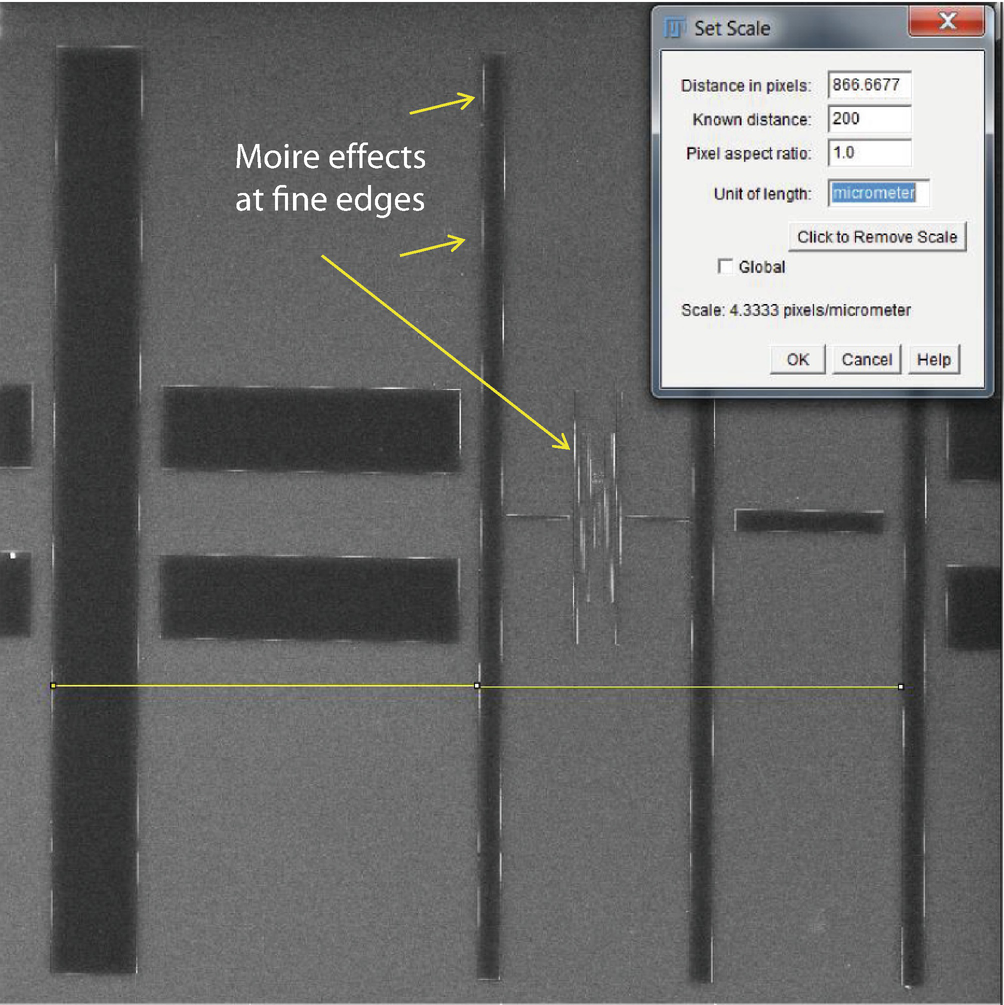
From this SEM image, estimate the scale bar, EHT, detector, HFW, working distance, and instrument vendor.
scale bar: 50000 nm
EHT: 20 kV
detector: SE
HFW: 233 µm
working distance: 17.02 mm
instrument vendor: Tescan